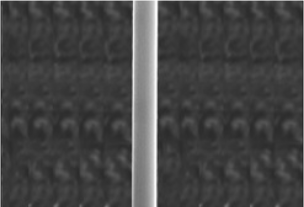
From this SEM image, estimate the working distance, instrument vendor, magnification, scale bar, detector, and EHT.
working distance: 8 mm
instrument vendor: Hitachi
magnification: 1 K X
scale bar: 50000 nm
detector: SE(M)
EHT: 15 kV